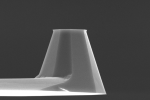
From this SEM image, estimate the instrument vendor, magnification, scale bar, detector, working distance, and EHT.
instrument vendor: JEOL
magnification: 2.7 K X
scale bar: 5000 nm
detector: SEI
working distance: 15 mm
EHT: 15 kV